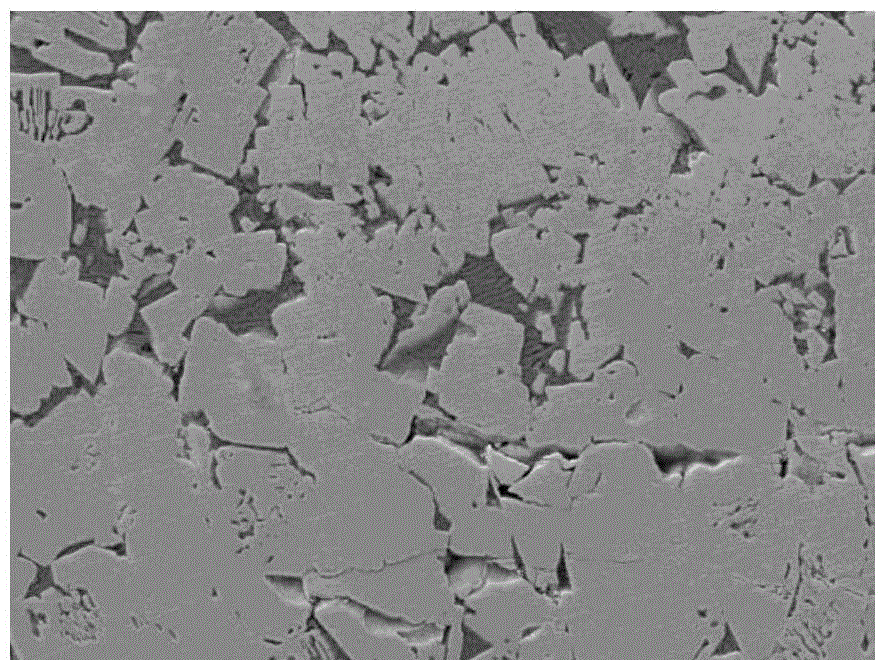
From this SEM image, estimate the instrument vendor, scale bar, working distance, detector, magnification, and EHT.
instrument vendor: JEOL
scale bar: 10000 nm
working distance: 15.2 mm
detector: LEI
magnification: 1 K X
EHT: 20 kV